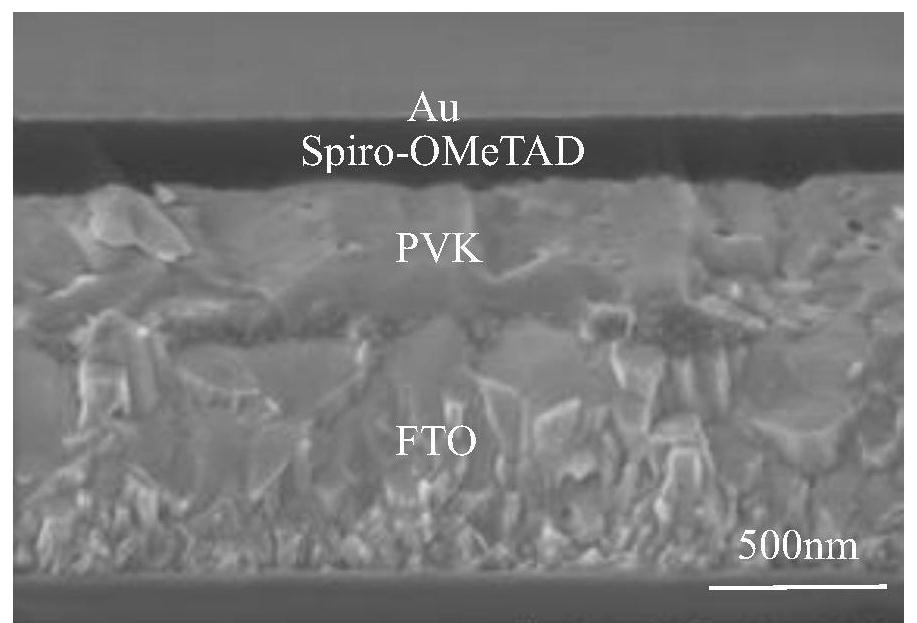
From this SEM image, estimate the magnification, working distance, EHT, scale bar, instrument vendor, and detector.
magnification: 50 K X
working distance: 12.6 mm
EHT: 3 kV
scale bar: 1000 nm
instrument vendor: Hitachi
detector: SE(TUL)